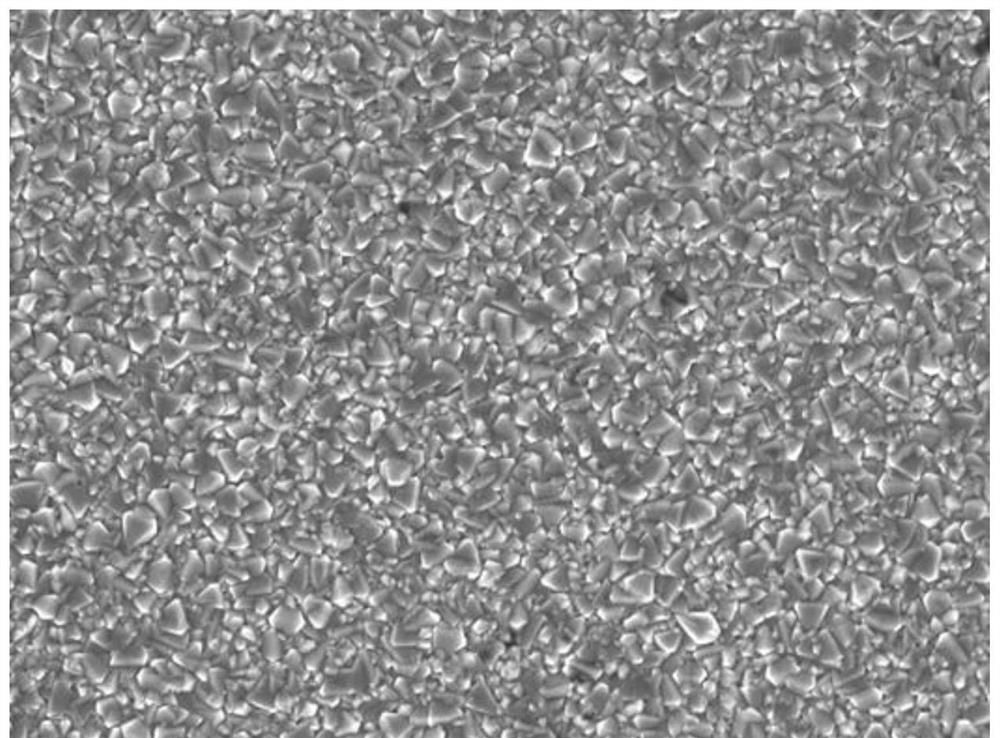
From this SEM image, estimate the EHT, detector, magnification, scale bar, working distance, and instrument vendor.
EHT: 5.5 kV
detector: SEI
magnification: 10 K X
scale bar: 1000 nm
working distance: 8.2 mm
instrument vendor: JEOL